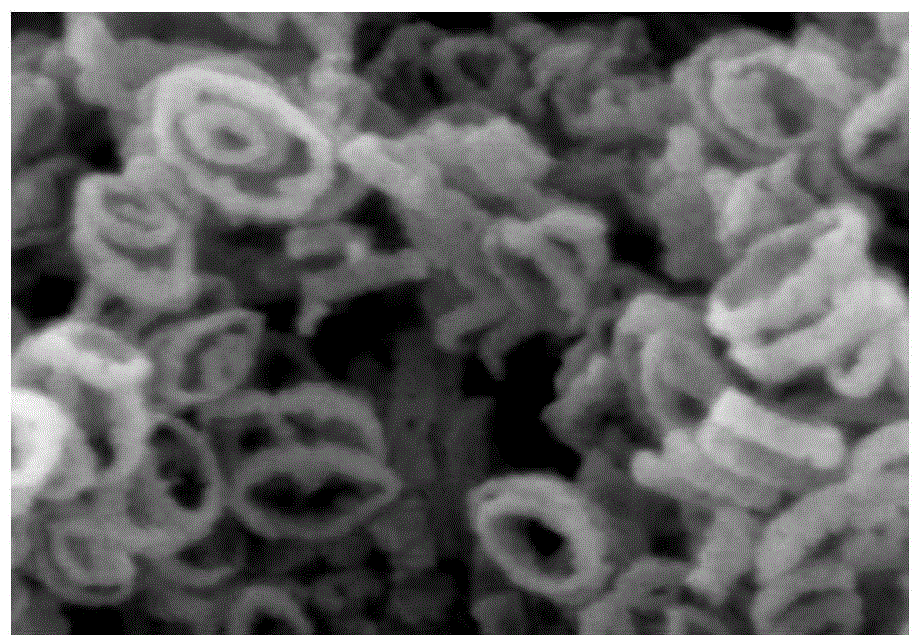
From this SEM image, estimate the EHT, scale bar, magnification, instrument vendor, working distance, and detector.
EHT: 5 kV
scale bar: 300 nm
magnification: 150 K X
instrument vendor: Hitachi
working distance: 6.9 mm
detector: SE(M)